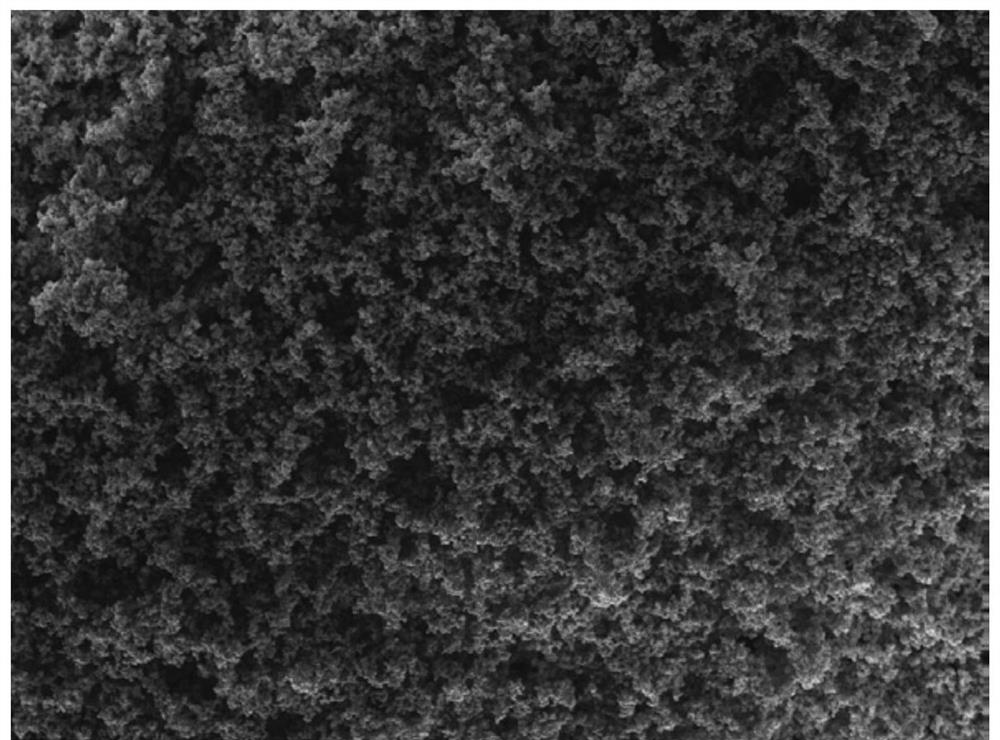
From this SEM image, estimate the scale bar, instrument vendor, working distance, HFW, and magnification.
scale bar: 500000 nm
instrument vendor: Tescan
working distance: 5.75 mm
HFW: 1860 µm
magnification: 0.15 K X